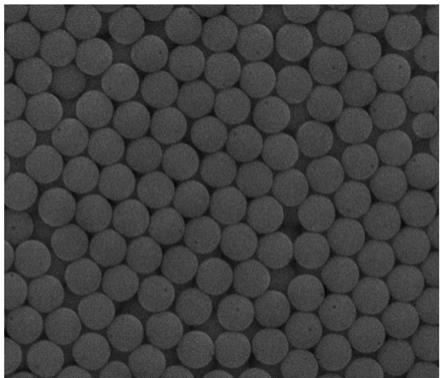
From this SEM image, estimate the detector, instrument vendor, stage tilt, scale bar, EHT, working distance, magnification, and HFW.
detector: ETD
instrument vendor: FEI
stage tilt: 0°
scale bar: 5000 nm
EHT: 30 kV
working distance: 10.8 mm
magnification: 20 K X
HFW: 14.9 µm